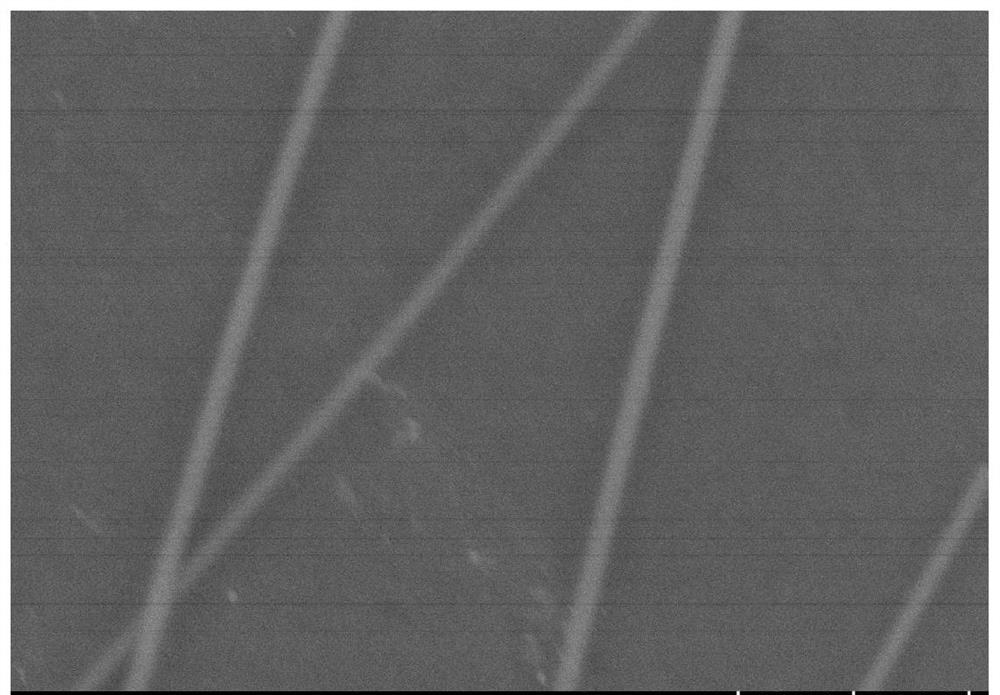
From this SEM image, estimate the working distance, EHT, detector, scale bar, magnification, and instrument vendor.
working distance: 8.2 mm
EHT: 5 kV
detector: SE(U)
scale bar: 500 nm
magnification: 60 K X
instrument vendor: Hitachi